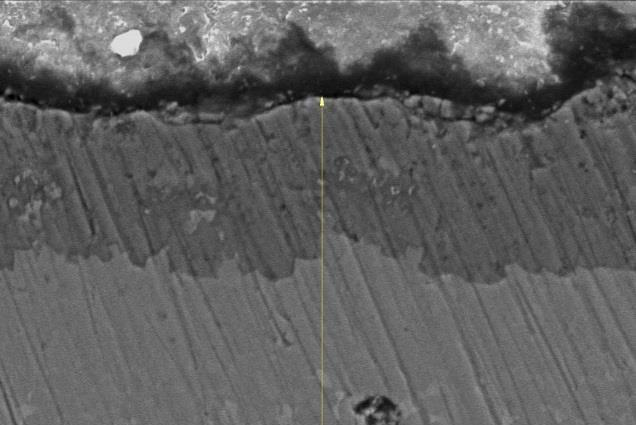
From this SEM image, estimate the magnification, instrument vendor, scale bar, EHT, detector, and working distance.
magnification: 1.5 K X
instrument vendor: other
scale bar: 20000 nm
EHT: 20 kV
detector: SE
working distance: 35 mm